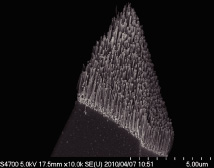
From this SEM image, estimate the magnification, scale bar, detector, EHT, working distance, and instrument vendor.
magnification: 10 K X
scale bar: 5000 nm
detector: SE(U)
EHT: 5 kV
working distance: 17.5 mm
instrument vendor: Hitachi